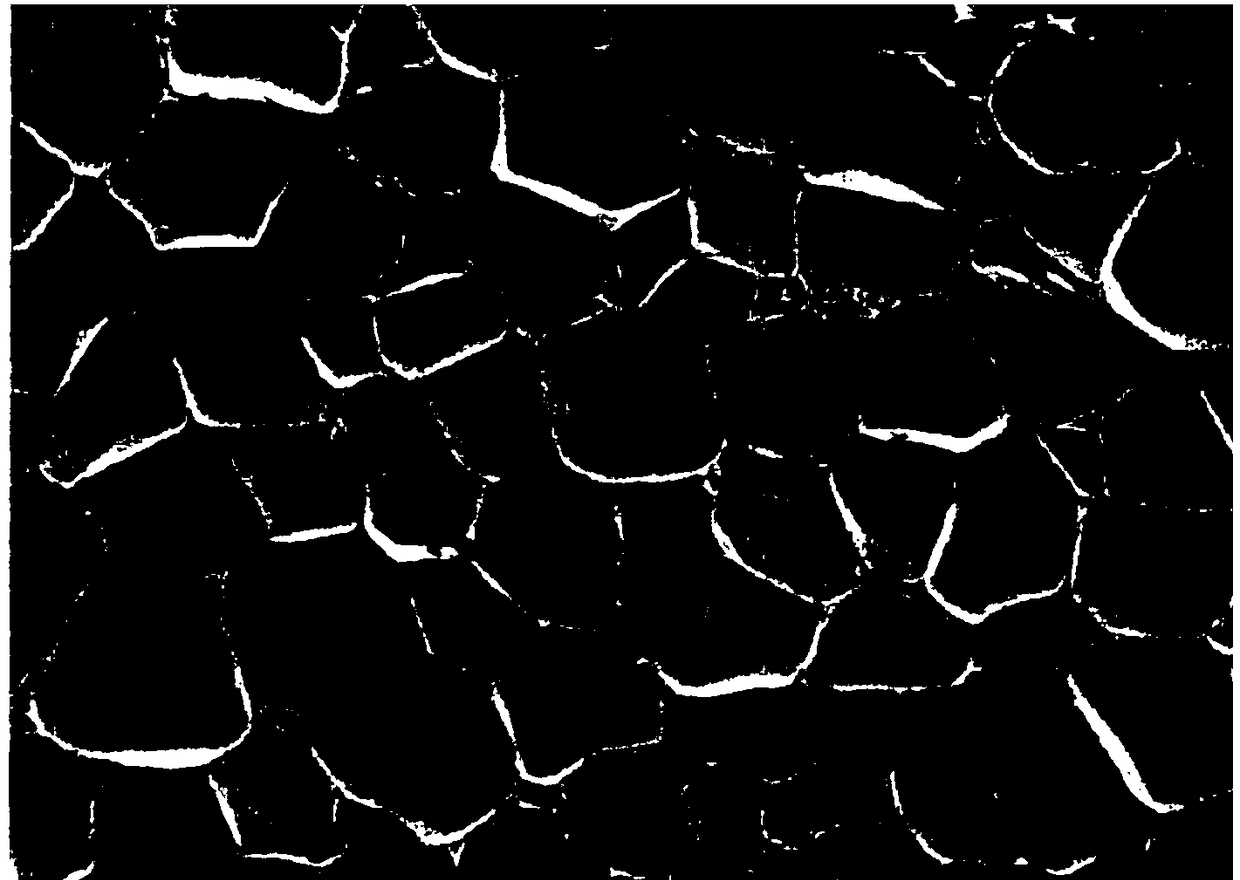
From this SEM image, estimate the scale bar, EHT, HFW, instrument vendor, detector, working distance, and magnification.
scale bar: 50000 nm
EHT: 5 kV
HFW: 277 µm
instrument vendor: Tescan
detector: SE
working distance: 7.56 mm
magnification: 1 K X